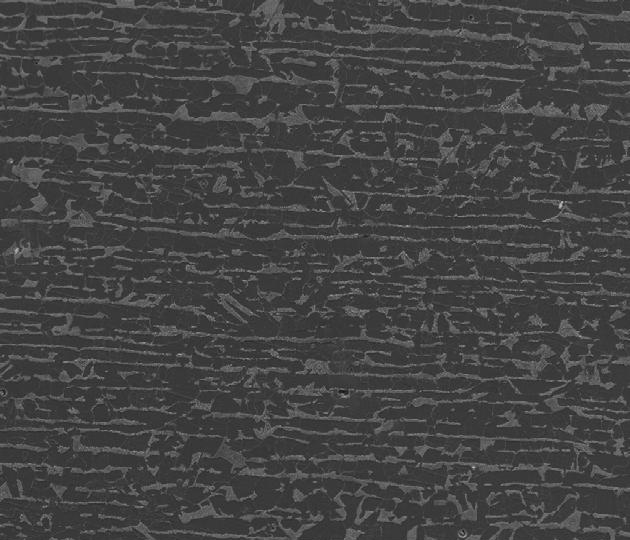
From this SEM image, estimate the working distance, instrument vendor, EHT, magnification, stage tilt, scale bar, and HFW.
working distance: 10 mm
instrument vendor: FEI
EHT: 25 kV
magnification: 0.5 K X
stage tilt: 1°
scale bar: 200000 nm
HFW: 541 µm